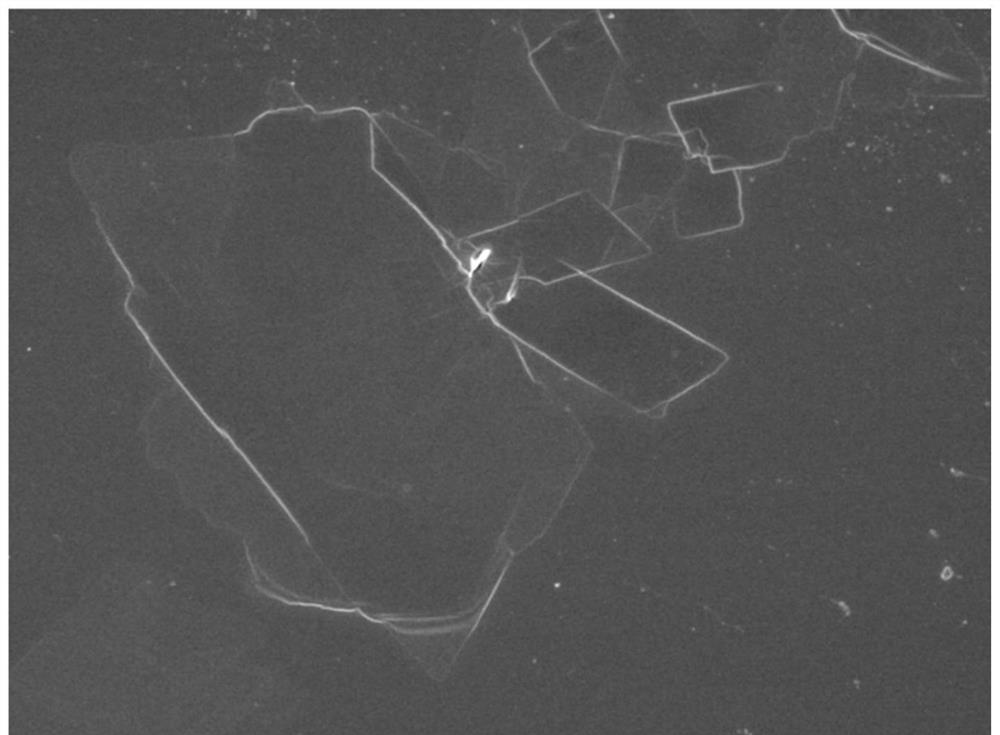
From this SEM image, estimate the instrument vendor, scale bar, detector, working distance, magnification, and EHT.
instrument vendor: JEOL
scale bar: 10000 nm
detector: SEI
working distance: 8.4 mm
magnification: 2.7 K X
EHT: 5 kV